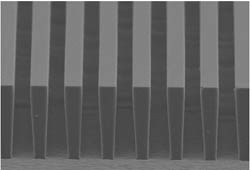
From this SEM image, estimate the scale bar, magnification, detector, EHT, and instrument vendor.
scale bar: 100000 nm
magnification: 0.35 K X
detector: SE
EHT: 15 kV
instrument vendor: Hitachi